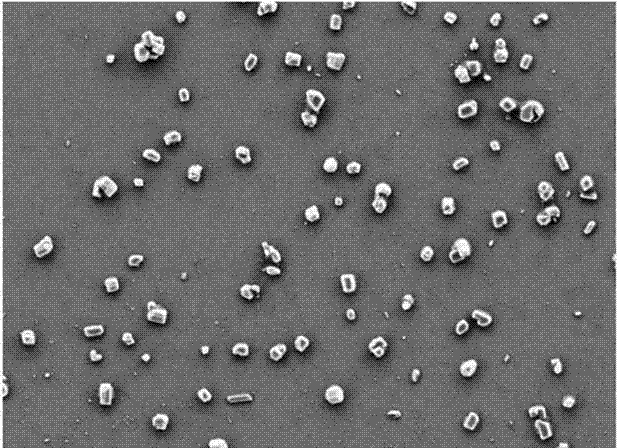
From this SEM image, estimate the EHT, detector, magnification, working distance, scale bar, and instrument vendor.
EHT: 3 kV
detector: SE2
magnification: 10 K X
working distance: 4.6 mm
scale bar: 2000 nm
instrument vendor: Zeiss